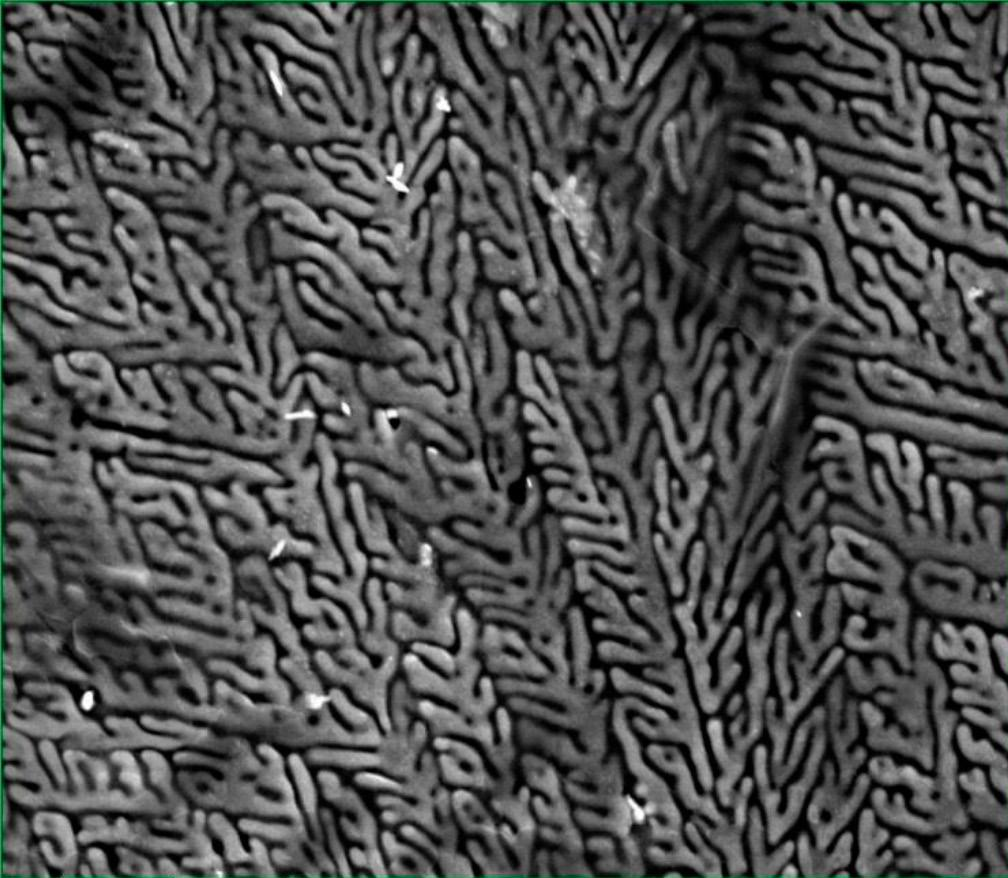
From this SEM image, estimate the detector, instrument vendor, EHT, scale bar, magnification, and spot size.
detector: ETD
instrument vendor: FEI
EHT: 10 kV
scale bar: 10000 nm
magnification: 12 K X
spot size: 3.5